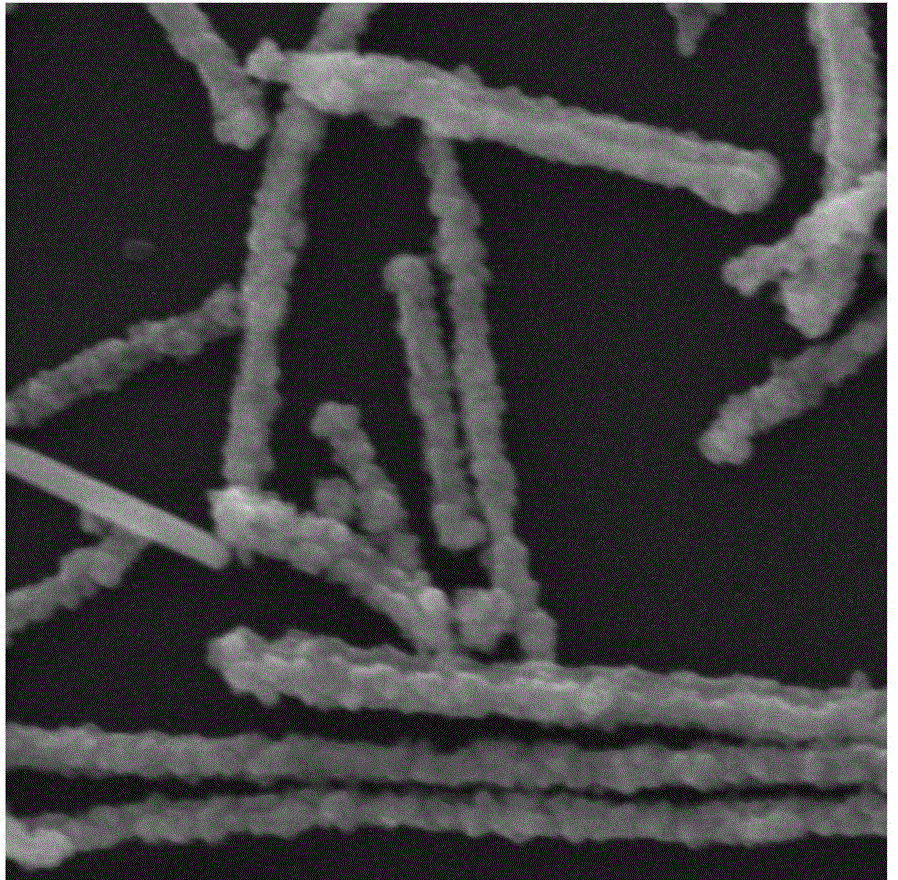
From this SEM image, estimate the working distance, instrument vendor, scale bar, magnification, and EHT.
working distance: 8.04 mm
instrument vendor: Tescan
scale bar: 2000 nm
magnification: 23.6 K X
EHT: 30 kV